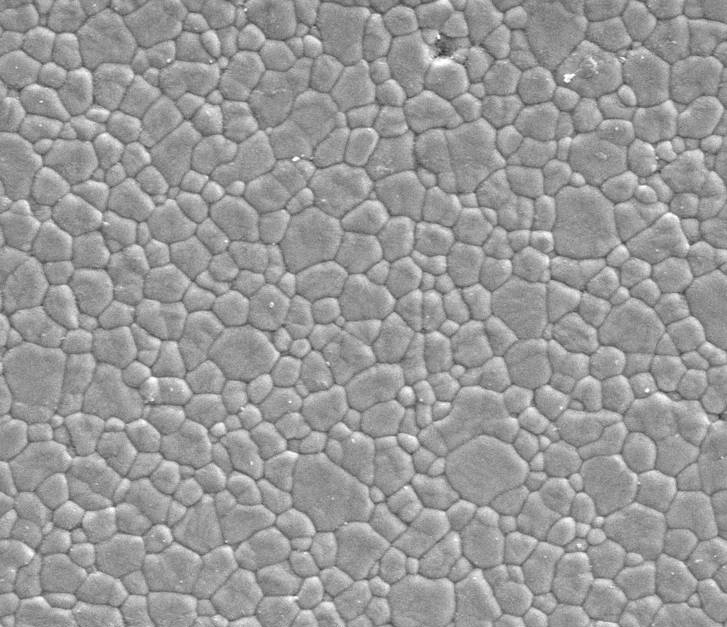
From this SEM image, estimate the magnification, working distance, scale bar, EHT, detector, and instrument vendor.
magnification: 10 K X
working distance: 5 mm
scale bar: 5000 nm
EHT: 5 kV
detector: ETD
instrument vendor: FEI